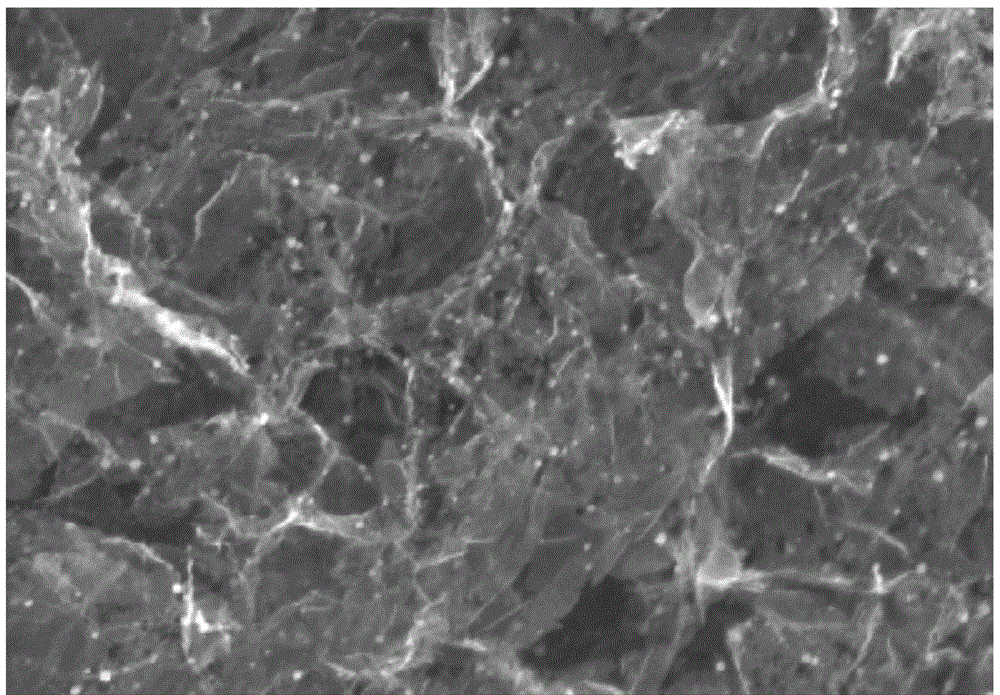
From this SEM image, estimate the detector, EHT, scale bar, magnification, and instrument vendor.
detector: SE(U)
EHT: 8 kV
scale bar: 1000 nm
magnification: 45 K X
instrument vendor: Hitachi